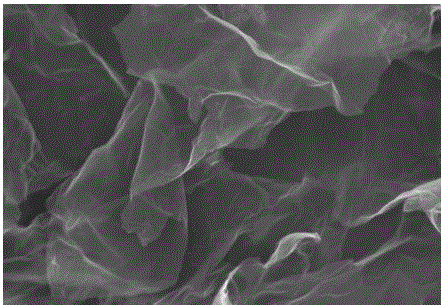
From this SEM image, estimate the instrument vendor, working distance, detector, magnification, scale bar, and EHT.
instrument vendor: Hitachi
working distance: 7.2 mm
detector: SE(M,LA0)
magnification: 50 K X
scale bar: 1000 nm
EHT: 5 kV